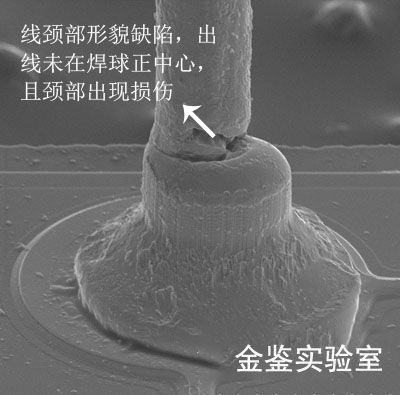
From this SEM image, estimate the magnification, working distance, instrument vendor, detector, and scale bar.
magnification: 0.8 K X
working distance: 20.6 mm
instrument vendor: Hitachi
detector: SE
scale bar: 50000 nm